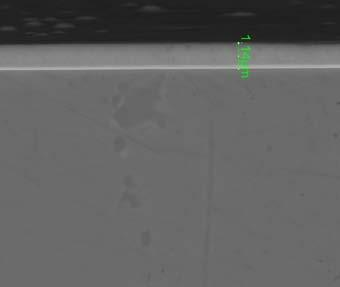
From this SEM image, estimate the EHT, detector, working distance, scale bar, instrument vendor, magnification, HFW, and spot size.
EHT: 20 kV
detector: BSED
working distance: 5.8 mm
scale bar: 5000 nm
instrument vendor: FEI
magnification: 10 K X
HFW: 14.9 µm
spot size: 4